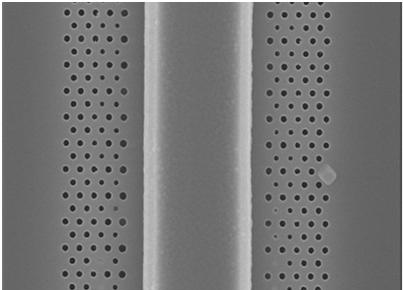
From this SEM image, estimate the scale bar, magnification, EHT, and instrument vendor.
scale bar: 2730 nm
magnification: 11 K X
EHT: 20 kV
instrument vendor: JEOL